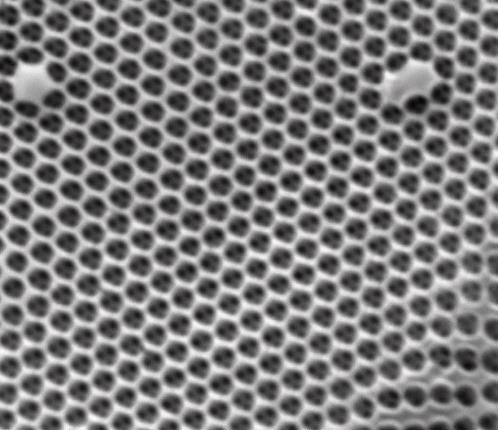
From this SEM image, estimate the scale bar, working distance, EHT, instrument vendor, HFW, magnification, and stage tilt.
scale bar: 2000 nm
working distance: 9.8 mm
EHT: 25 kV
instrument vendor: FEI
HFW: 5.41 µm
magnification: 50 K X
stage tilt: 0°